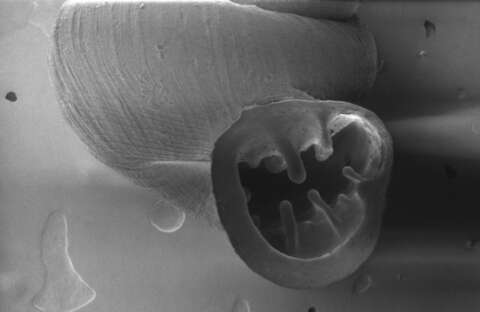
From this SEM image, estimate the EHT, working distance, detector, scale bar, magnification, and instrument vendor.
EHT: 1 kV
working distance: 30.5 mm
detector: SE1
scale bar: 200000 nm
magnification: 0.05 K X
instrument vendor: Zeiss